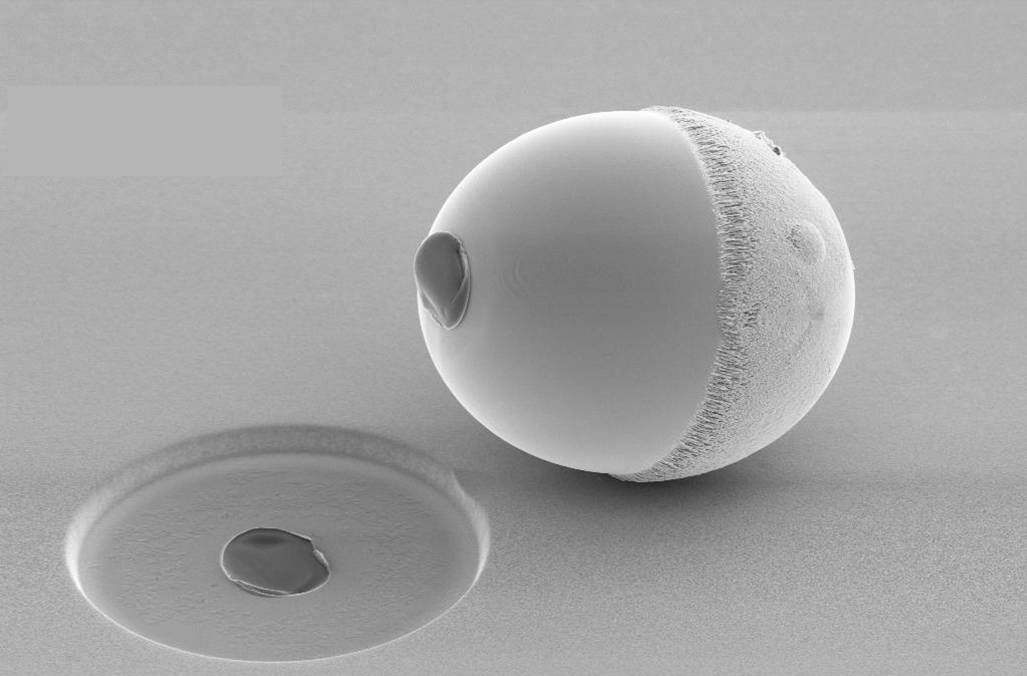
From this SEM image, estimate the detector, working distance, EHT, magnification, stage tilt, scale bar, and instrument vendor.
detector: SE2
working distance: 17 mm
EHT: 5 kV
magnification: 2.12 K X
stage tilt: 52.2°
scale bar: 3000 nm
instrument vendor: Zeiss